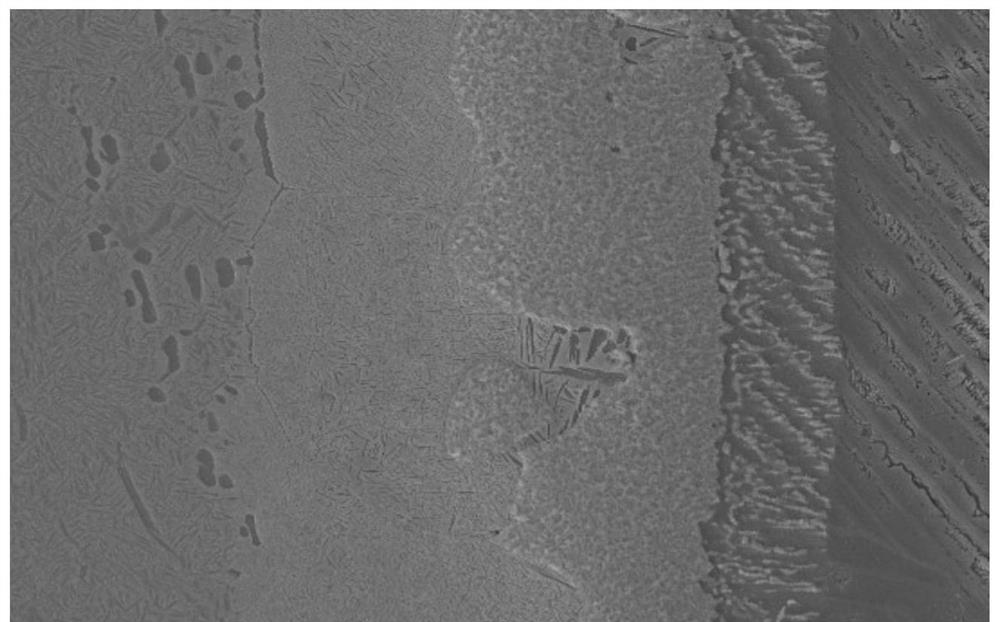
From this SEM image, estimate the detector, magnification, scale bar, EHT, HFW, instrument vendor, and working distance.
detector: SE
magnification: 2.5 K X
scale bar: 20000 nm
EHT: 20 kV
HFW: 111 µm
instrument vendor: Tescan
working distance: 13.98 mm